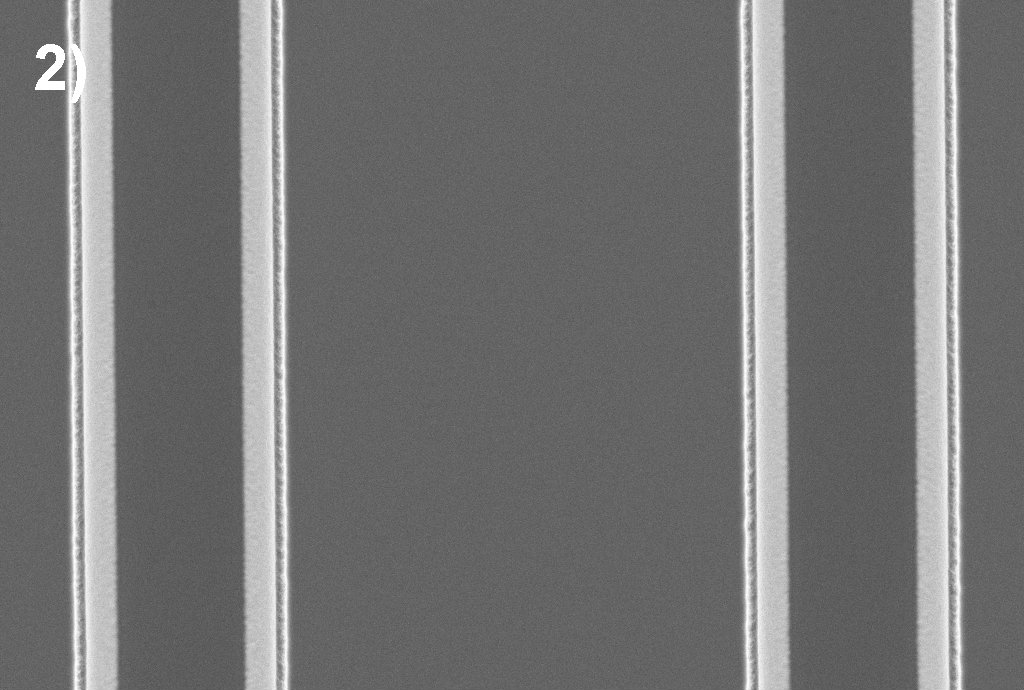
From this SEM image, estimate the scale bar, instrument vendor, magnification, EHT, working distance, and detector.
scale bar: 2000 nm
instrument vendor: Zeiss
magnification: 2.5 K X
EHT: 5 kV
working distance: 4.5 mm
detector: InLens